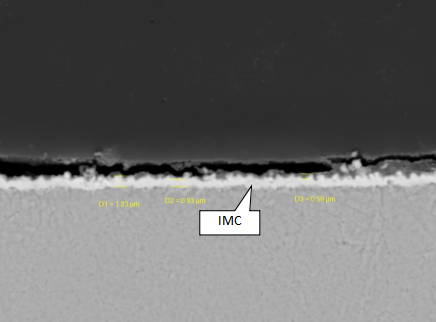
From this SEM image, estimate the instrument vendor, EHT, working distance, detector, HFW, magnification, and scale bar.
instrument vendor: Tescan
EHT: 20 kV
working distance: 15.13 mm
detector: BSE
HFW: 50.3 µm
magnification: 5.5 K X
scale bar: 10000 nm